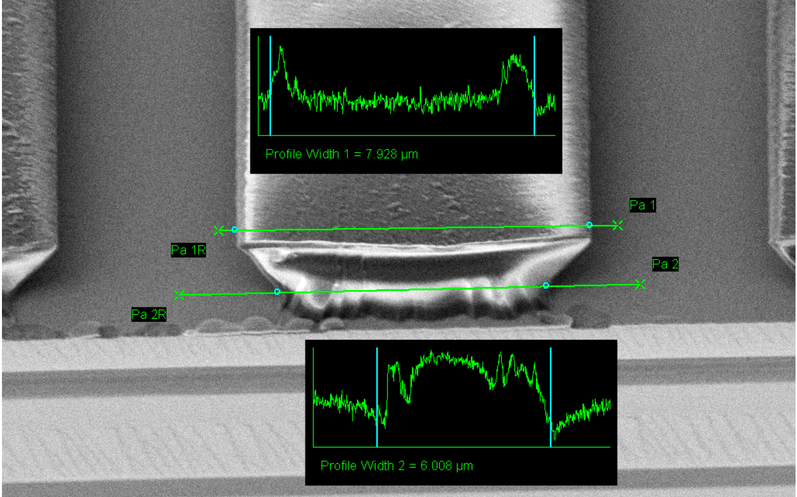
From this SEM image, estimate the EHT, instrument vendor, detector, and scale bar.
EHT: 2 kV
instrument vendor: Zeiss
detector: SE2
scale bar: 2000 nm